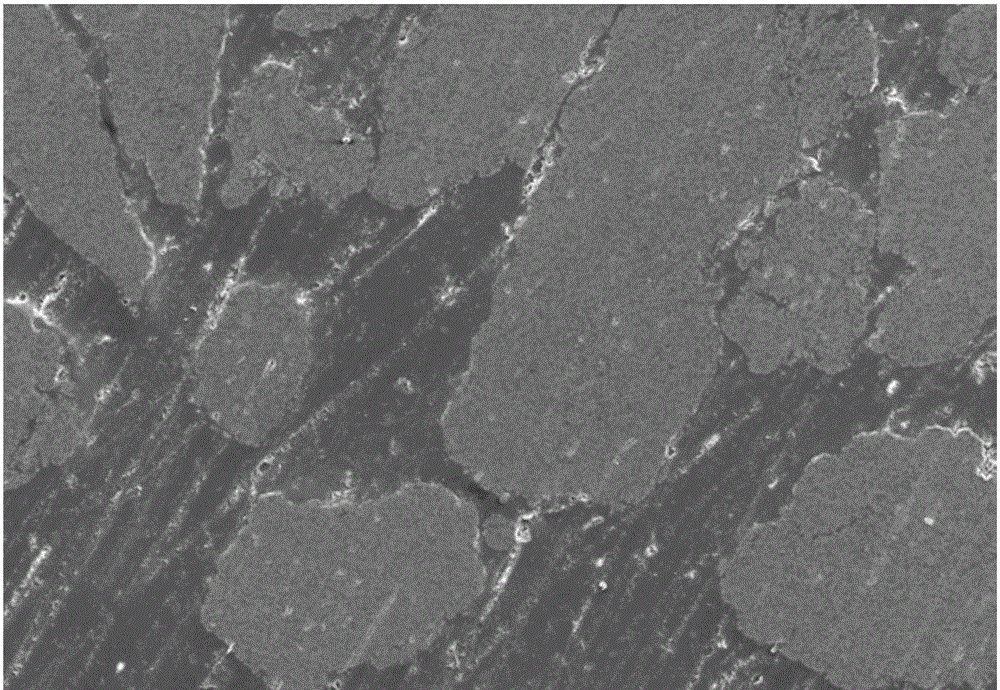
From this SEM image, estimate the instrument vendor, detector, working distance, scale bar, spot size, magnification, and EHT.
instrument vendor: JEOL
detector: SEI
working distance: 12 mm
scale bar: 100000 nm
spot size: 58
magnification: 0.1 K X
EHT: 20 kV